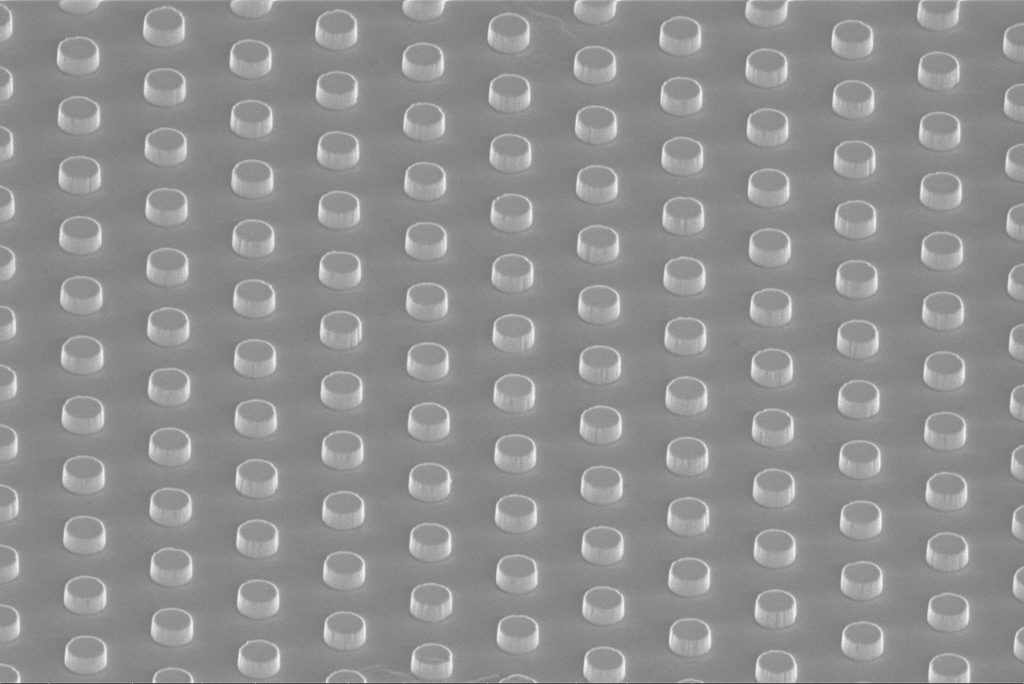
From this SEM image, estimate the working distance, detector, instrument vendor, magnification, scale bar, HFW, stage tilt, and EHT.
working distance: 3.8 mm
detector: TLD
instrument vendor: FEI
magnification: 10 K X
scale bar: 5000 nm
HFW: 20.7 µm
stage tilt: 52°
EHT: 2 kV